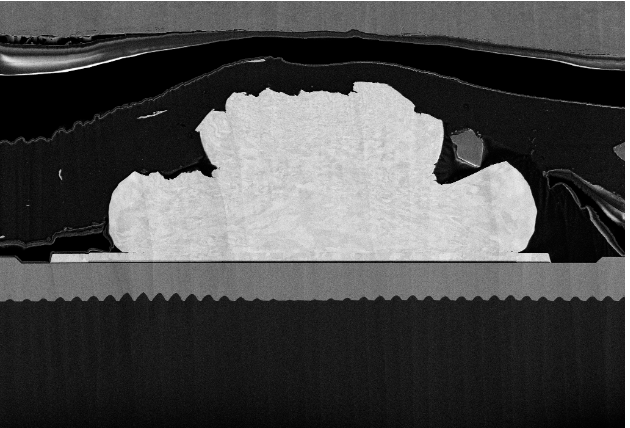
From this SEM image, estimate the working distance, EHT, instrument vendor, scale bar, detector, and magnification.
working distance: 4.9 mm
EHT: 5 kV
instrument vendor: Zeiss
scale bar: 10000 nm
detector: SE2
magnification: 1 K X